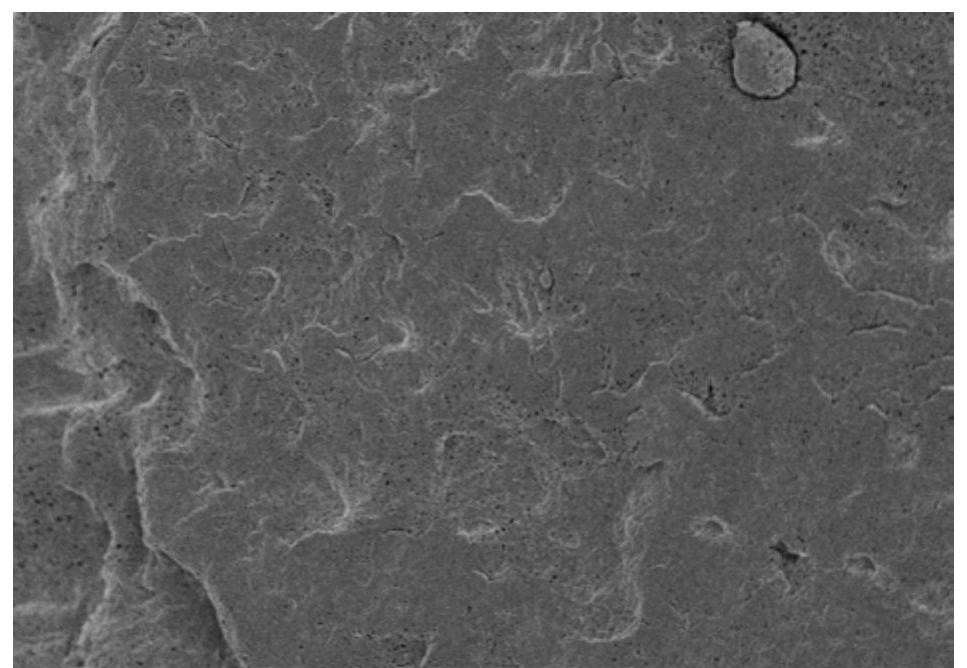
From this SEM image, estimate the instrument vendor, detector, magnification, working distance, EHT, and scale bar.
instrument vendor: Hitachi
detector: SE(M)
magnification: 2.5 K X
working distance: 7 mm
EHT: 3 kV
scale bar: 20000 nm